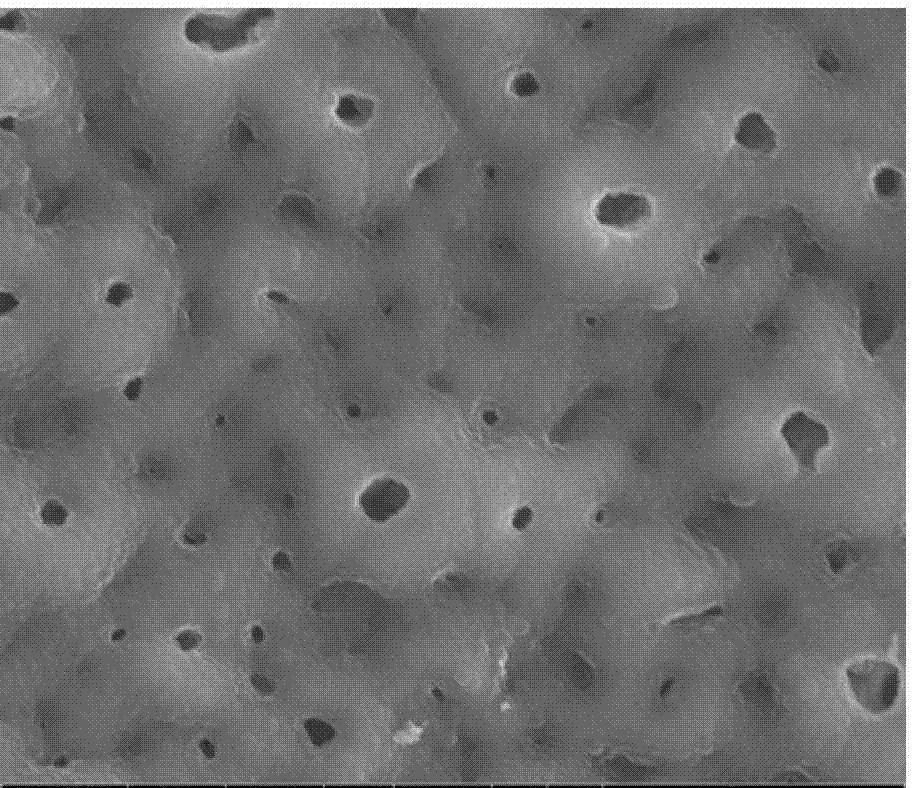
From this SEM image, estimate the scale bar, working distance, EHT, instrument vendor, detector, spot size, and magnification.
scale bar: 5000 nm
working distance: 10.5 mm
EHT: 20 kV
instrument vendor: FEI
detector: ETD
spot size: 3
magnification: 10 K X